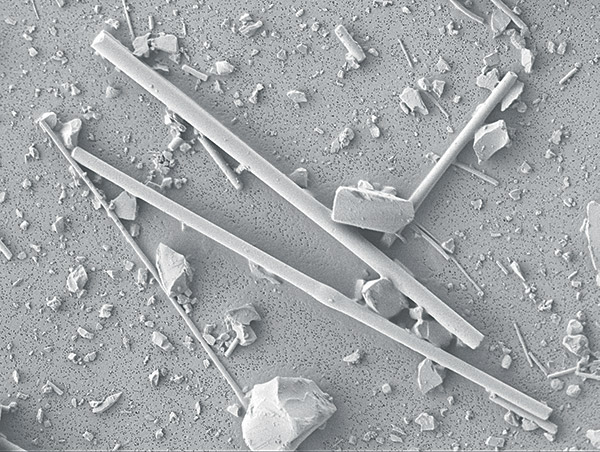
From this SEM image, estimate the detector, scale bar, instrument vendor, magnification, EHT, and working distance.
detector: LEI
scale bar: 10000 nm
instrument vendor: JEOL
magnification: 0.6 K X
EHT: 2 kV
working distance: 6.8 mm